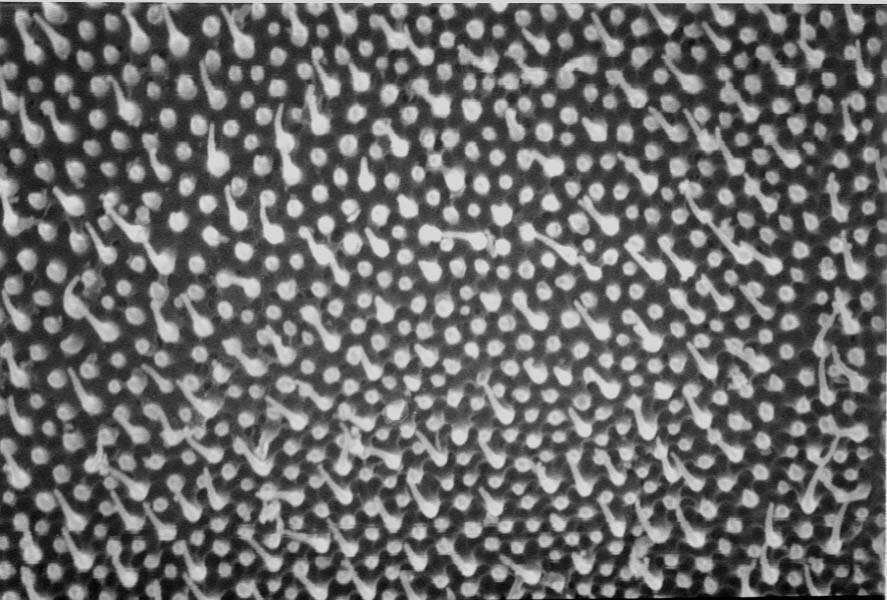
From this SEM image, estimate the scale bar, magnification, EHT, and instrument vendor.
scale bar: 1000 nm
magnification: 10 K X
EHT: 20 kV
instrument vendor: JEOL